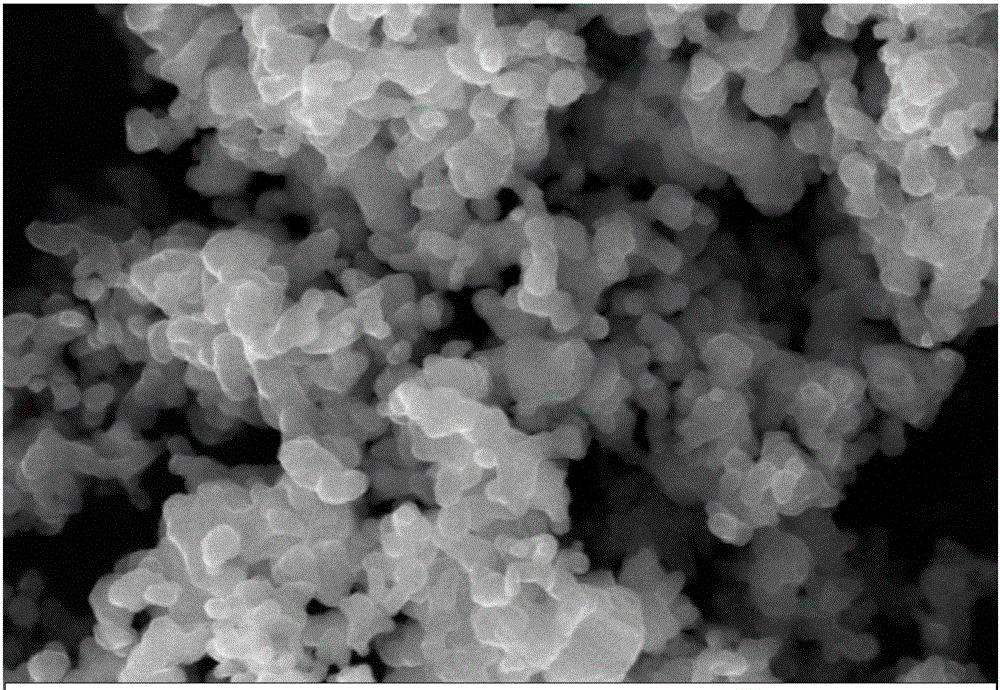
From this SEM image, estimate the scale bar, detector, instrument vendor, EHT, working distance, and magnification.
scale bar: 200 nm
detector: SE1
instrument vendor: Zeiss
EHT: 20 kV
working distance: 8 mm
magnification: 20 K X